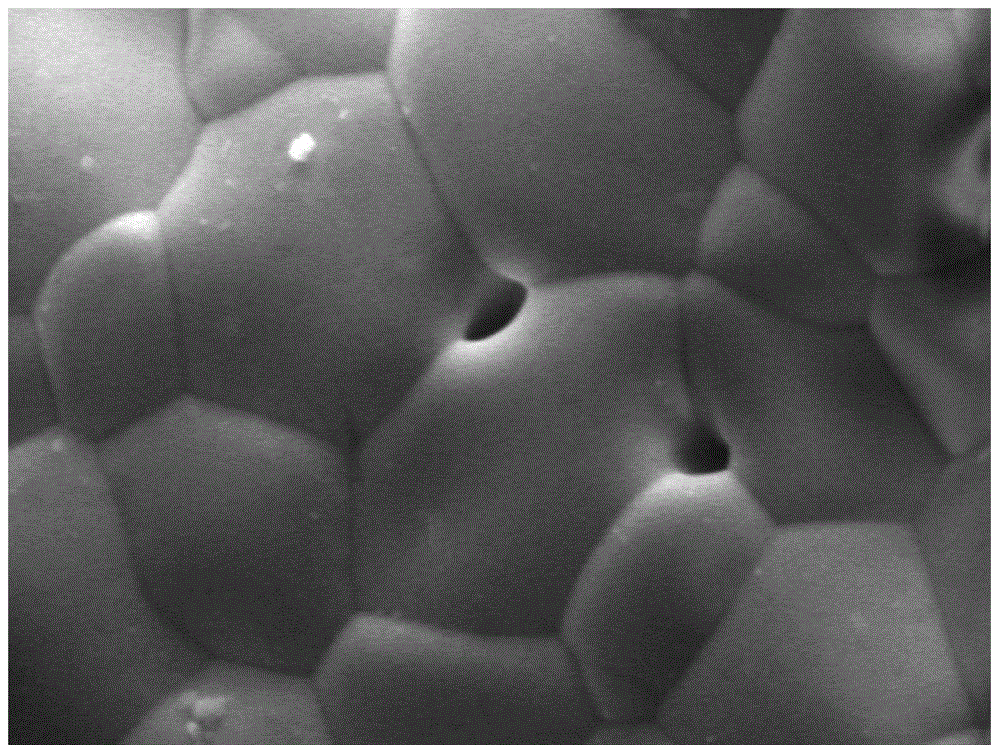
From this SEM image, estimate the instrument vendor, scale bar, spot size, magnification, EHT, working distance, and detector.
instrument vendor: FEI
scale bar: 2000 nm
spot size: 5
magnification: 10 K X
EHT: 10 kV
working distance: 5.6 mm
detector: SE